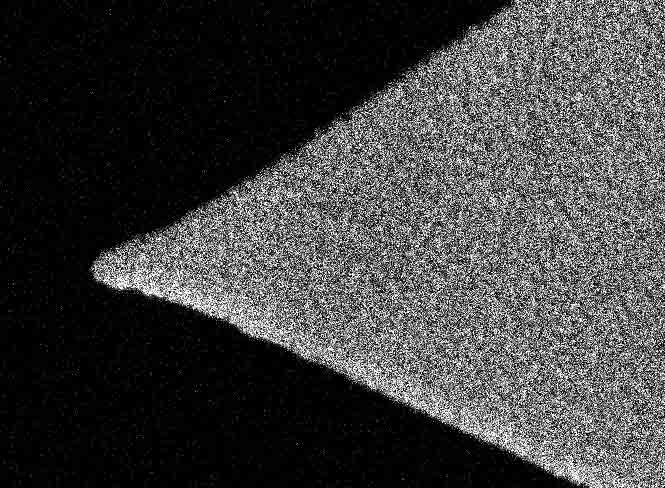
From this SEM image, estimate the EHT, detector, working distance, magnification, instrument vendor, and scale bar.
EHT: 5 kV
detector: SEI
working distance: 5.4 mm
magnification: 100 K X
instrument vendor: JEOL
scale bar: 100 nm